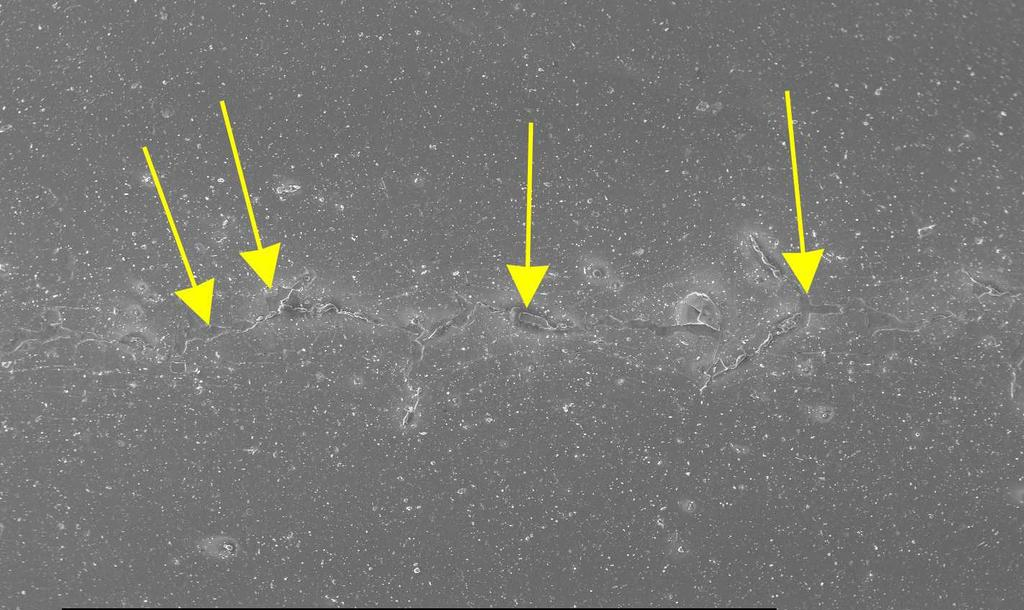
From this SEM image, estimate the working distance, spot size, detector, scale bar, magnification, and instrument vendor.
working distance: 11.2 mm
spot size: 5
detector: SE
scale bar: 500000 nm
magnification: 0.05 K X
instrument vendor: FEI